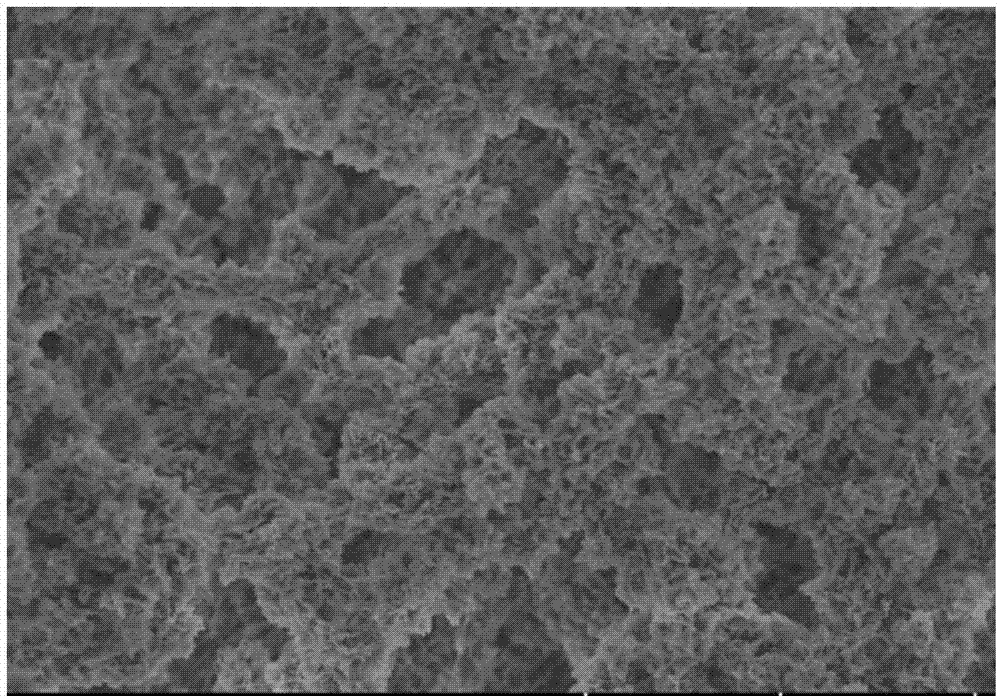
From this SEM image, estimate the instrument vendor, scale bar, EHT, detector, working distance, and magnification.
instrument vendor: Hitachi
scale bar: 5000 nm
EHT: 3 kV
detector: SE(UL)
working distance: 7.9 mm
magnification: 10 K X